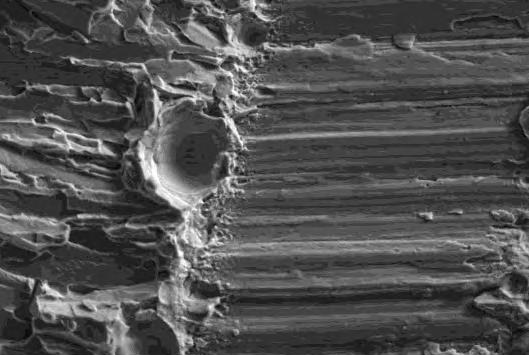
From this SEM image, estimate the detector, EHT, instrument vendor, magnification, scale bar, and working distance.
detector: SE1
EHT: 20 kV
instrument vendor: Zeiss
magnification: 2 K X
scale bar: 10000 nm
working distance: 8.5 mm